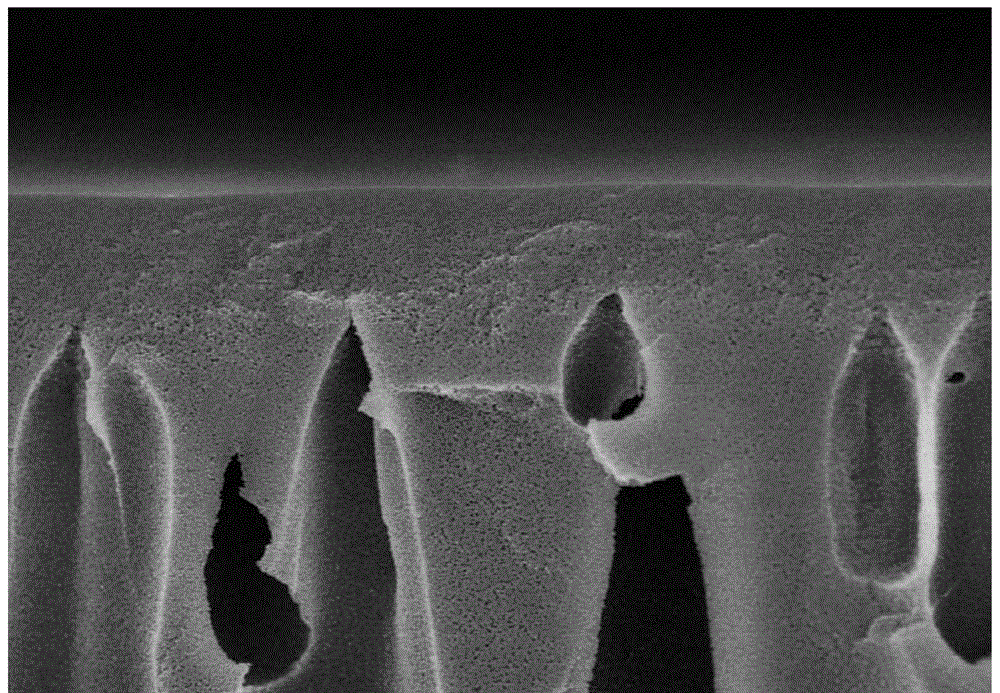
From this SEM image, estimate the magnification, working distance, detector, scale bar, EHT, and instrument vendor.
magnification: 5 K X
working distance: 2.9 mm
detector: SE(U)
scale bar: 10000 nm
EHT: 5 kV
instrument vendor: Hitachi